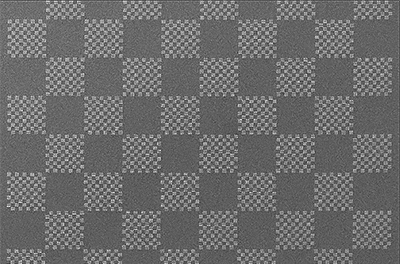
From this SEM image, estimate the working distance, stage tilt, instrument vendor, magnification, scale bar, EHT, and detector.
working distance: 4.3 mm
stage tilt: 0°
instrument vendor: FEI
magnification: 0.12 K X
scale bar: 400000 nm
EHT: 5 kV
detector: ETD